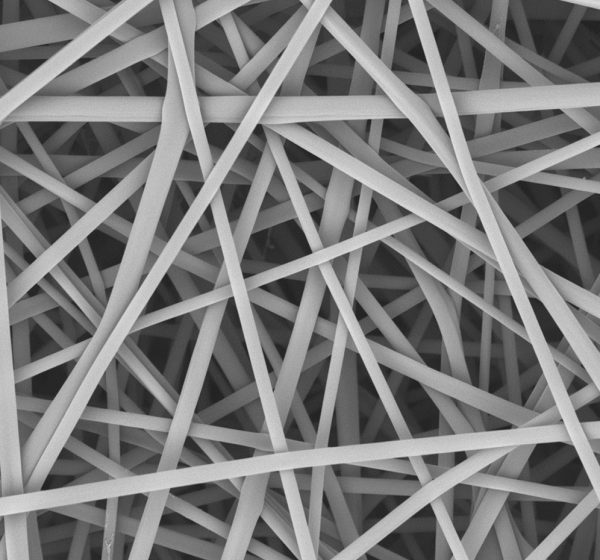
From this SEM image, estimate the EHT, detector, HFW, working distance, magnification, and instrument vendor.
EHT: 10 kV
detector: BSD Full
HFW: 59.8 µm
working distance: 6.83 mm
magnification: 10 K X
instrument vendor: other